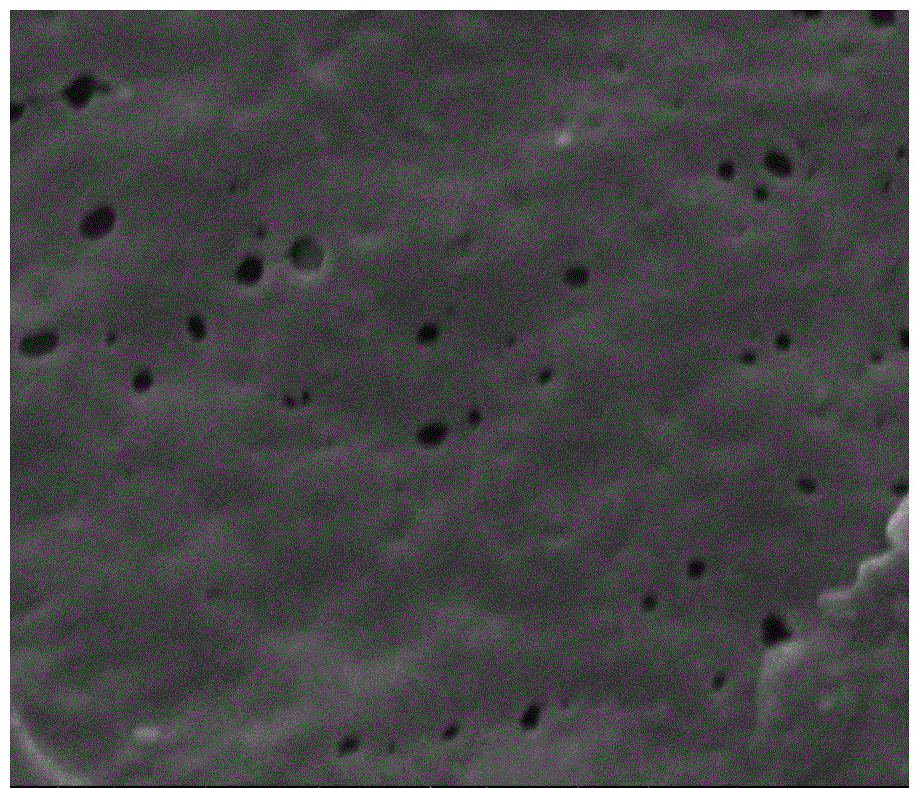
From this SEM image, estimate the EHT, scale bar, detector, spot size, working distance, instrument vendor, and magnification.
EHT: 10 kV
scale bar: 500 nm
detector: ETD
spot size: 3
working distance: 4.1 mm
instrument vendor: FEI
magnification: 100 K X